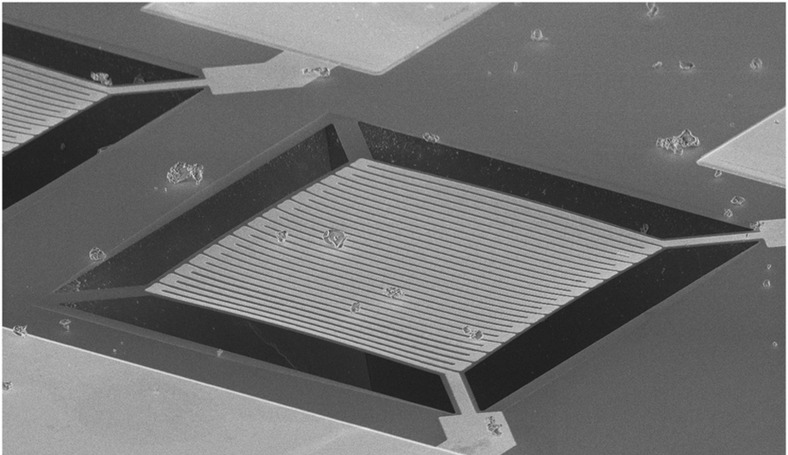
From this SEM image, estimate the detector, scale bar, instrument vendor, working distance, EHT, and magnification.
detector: SEI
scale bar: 100000 nm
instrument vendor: JEOL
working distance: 22.4 mm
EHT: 10 kV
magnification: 0.2 K X